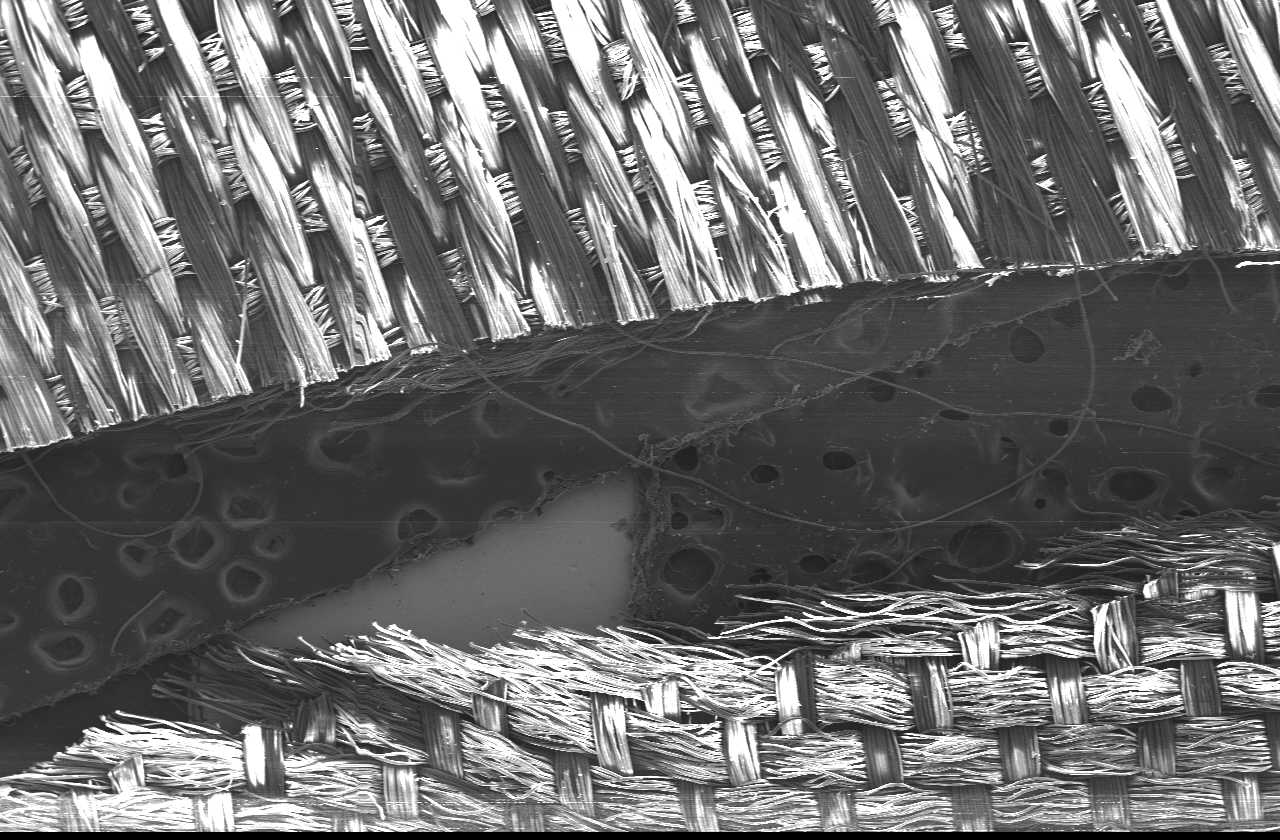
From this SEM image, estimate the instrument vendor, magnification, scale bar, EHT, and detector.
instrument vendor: JEOL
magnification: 0.02 K X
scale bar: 1000000 nm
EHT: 15 kV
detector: SEI